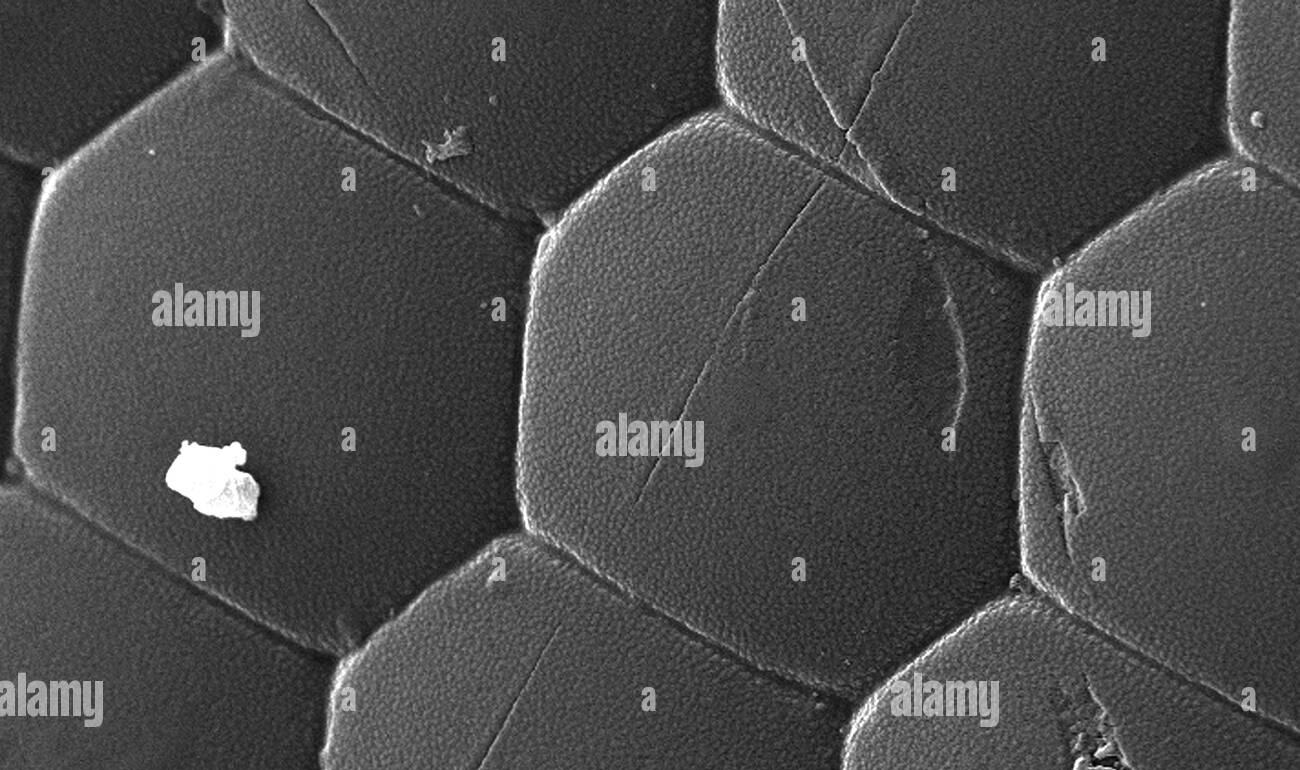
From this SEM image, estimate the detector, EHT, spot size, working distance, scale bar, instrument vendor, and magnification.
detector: SE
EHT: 30 kV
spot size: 3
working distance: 7.7 mm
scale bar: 10000 nm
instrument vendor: FEI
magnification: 2.917 K X